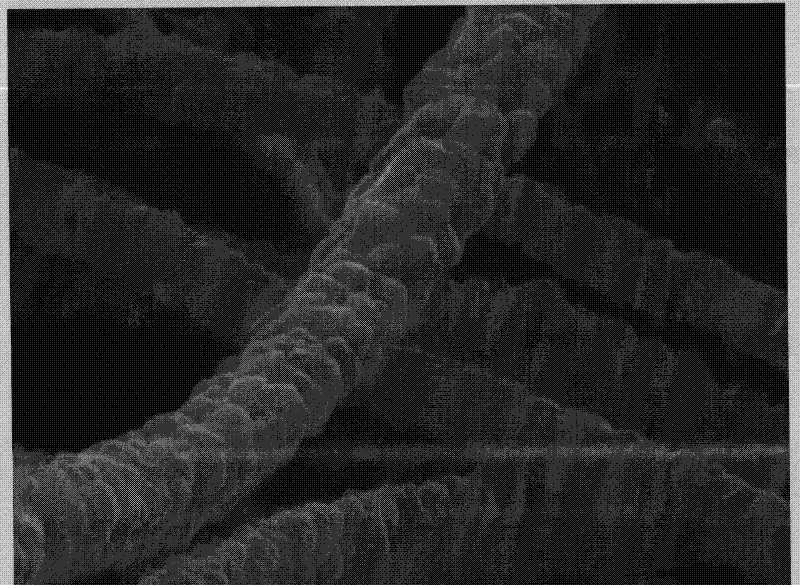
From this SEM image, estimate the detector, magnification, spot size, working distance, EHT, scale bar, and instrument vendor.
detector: SE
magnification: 40 K X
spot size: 5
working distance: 9.7 mm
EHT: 20 kV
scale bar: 500 nm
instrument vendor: FEI